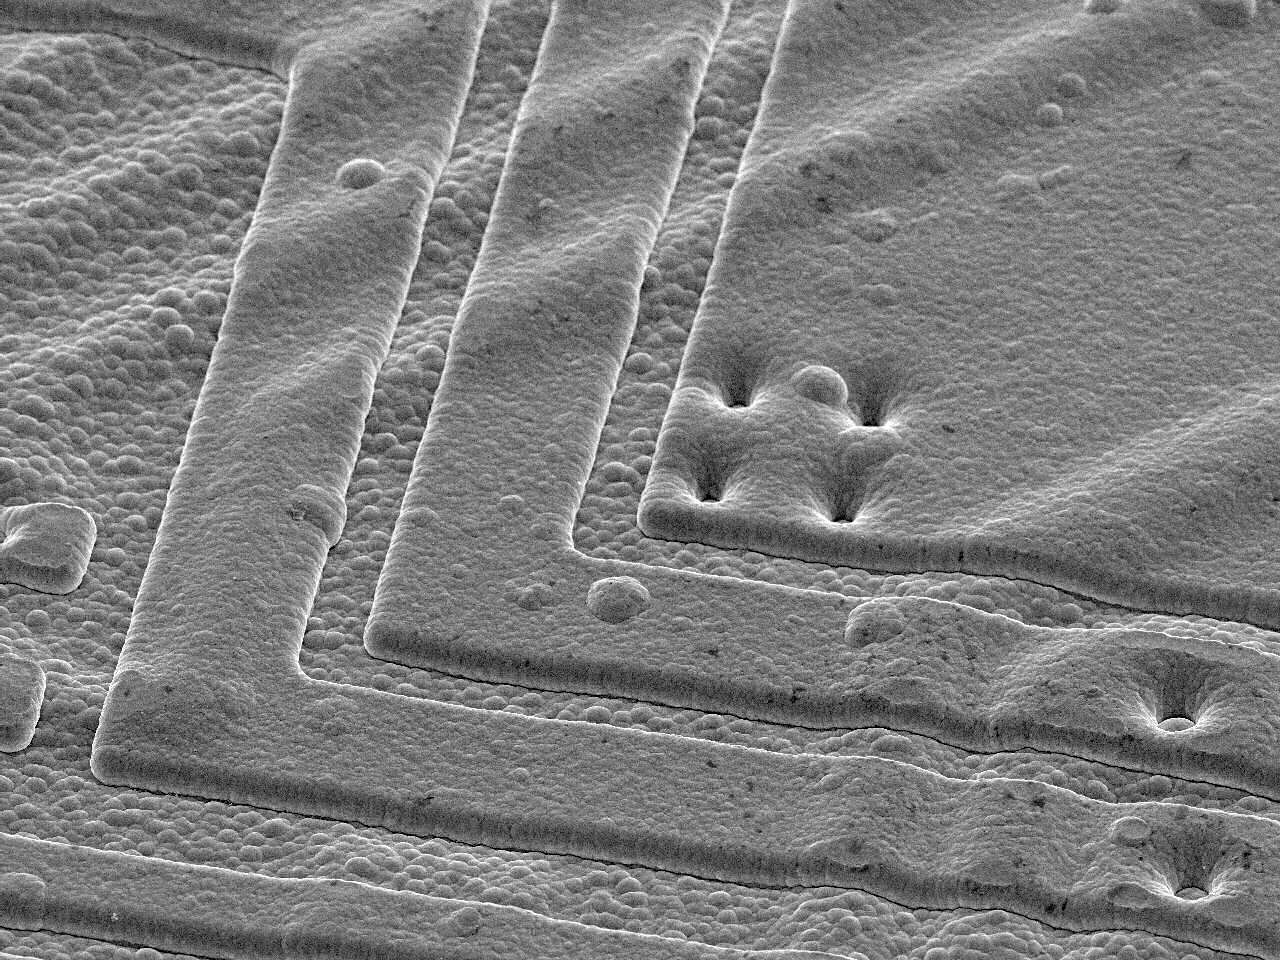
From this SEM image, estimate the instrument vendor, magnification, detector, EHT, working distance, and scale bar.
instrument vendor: JEOL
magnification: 2 K X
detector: SEI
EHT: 5 kV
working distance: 10 mm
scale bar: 10000 nm